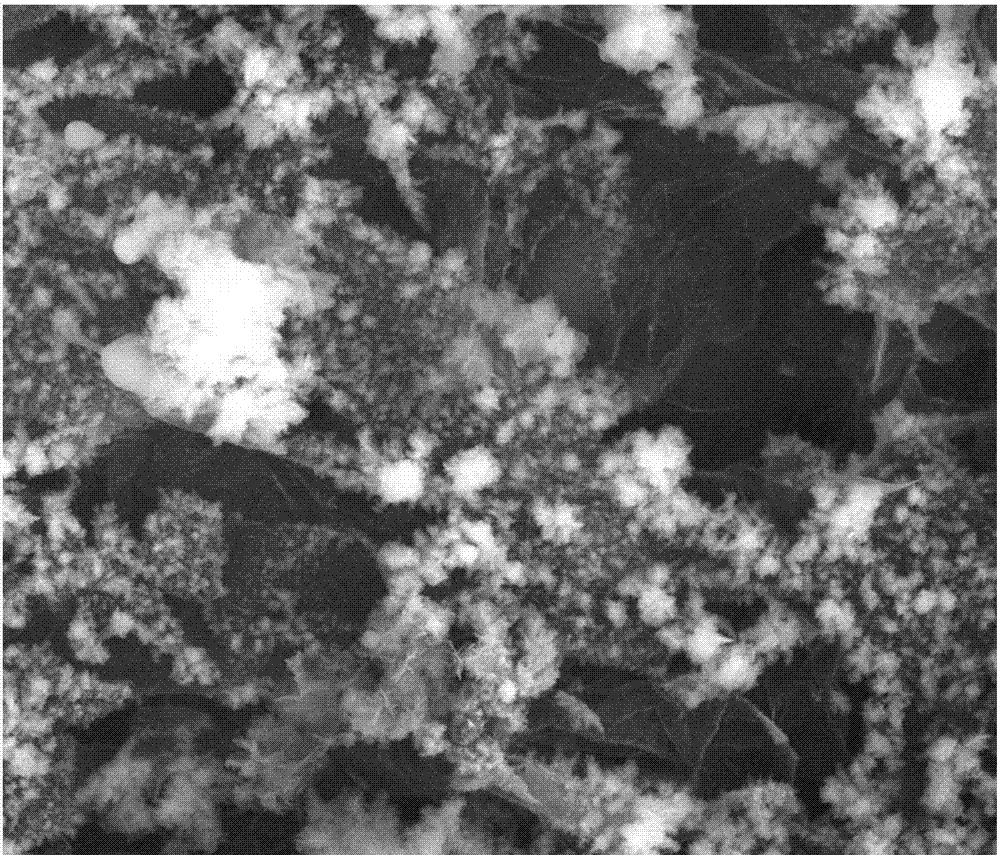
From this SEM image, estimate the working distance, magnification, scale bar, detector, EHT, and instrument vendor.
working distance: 2.5 mm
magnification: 10 K X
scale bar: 5000 nm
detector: SE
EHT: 20 kV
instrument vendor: FEI